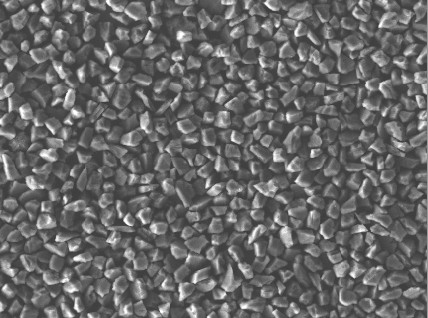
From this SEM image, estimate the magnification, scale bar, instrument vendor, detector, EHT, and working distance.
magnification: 1 K X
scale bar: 10000 nm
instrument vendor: JEOL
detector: LEI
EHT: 15 kV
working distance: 9.7 mm